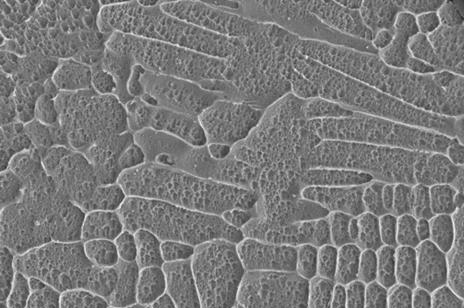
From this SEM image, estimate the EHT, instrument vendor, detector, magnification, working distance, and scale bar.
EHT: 5 kV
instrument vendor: Zeiss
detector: SE2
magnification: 5 K X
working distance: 16.9 mm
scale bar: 2000 nm